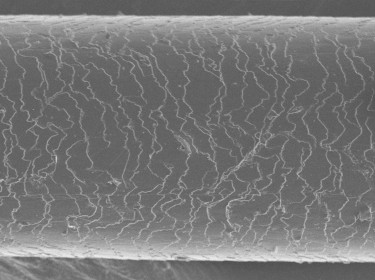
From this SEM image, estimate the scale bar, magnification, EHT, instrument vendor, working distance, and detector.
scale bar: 10000 nm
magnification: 0.75 K X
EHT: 5 kV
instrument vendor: JEOL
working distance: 11 mm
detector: SEI